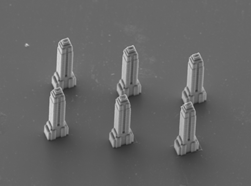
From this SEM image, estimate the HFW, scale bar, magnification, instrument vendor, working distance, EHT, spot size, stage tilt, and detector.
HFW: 298 µm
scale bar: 100000 nm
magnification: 1 K X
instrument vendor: FEI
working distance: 10.2 mm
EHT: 15 kV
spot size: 4.5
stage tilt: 24°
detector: ETD